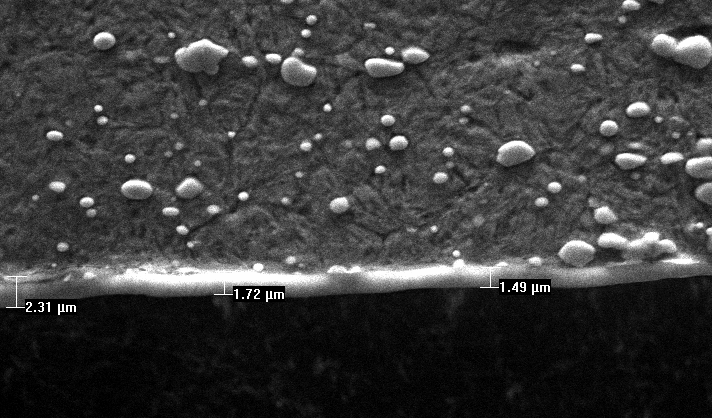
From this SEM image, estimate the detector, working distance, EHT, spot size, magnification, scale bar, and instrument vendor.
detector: SE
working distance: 11.6 mm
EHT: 20 kV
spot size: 4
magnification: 2 K X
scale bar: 10000 nm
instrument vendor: FEI